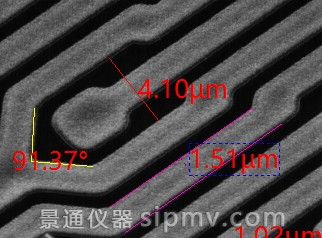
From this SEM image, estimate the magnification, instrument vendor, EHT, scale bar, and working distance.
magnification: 5 K X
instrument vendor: JEOL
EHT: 25 kV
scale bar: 1000 nm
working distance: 8 mm